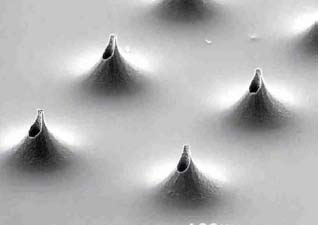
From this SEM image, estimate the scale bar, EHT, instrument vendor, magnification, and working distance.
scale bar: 100000 nm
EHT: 25 kV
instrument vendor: JEOL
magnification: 0.08 K X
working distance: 21 mm